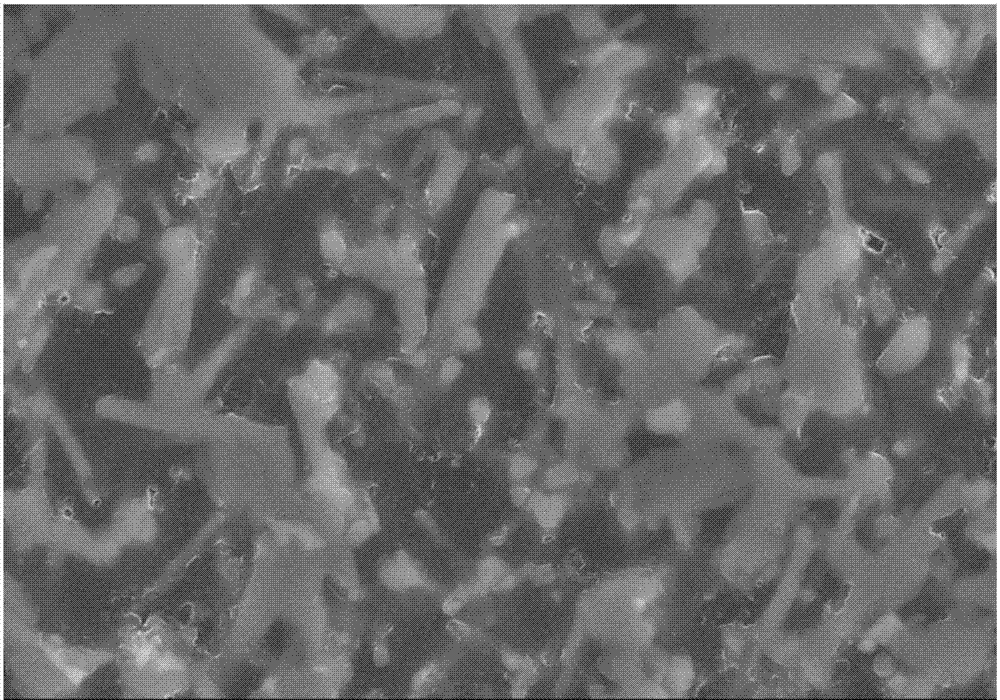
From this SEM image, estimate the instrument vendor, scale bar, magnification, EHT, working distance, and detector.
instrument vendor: Hitachi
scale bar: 5000 nm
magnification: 10 K X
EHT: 10 kV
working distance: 8.6 mm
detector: SE(M)